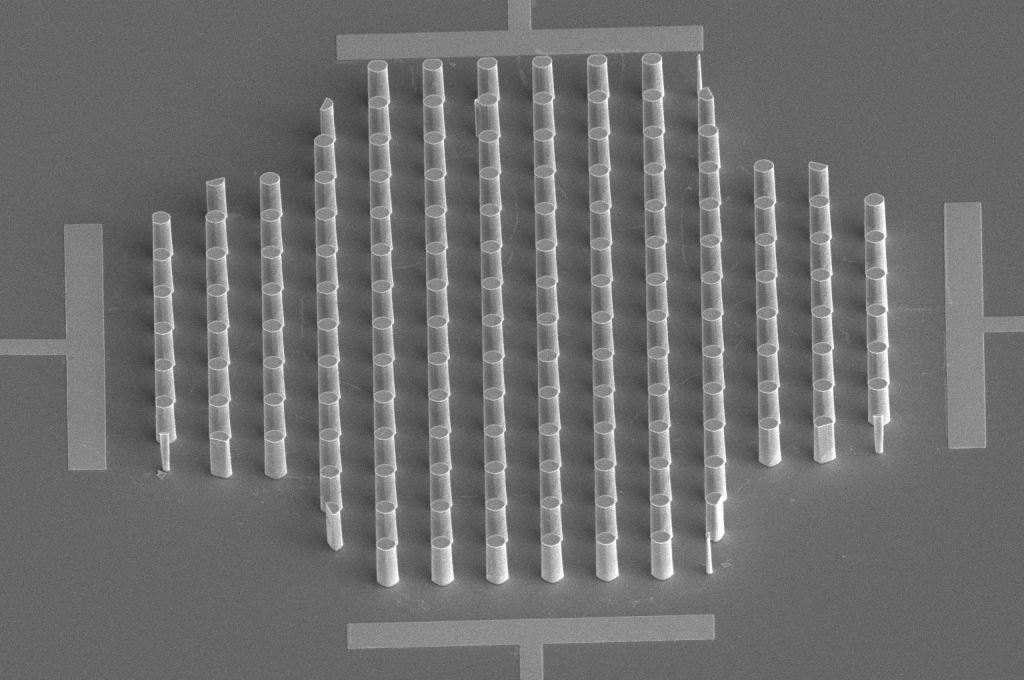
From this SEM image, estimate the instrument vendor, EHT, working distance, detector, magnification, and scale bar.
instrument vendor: FEI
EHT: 5 kV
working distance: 23.4 mm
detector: ETD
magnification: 1.5 K X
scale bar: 50000 nm